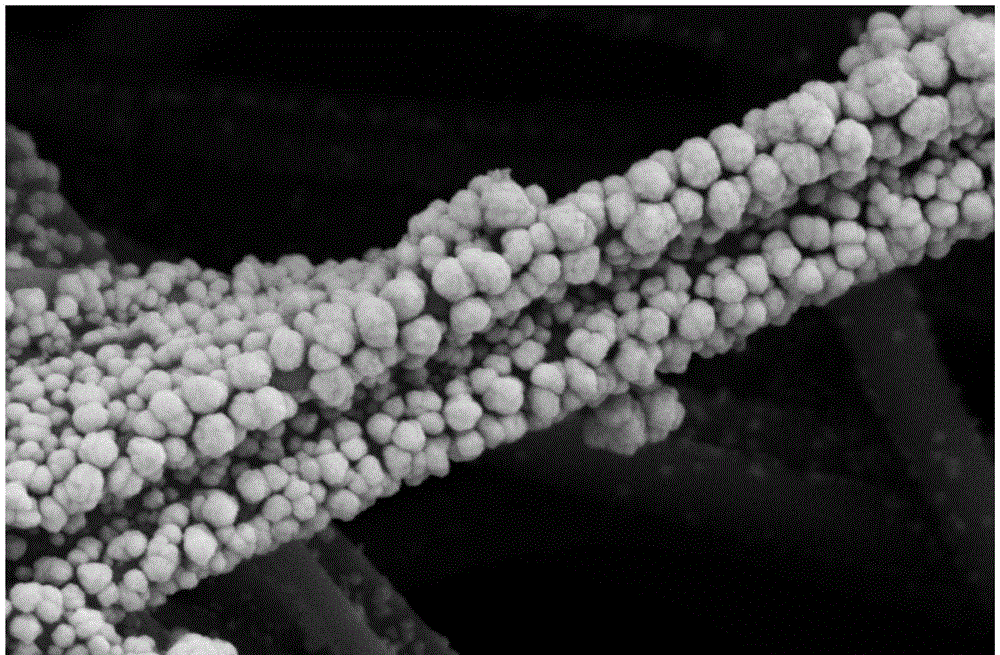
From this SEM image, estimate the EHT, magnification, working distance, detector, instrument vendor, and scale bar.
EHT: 5 kV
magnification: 50 K X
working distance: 6.8 mm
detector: SE2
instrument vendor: Zeiss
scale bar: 200 nm